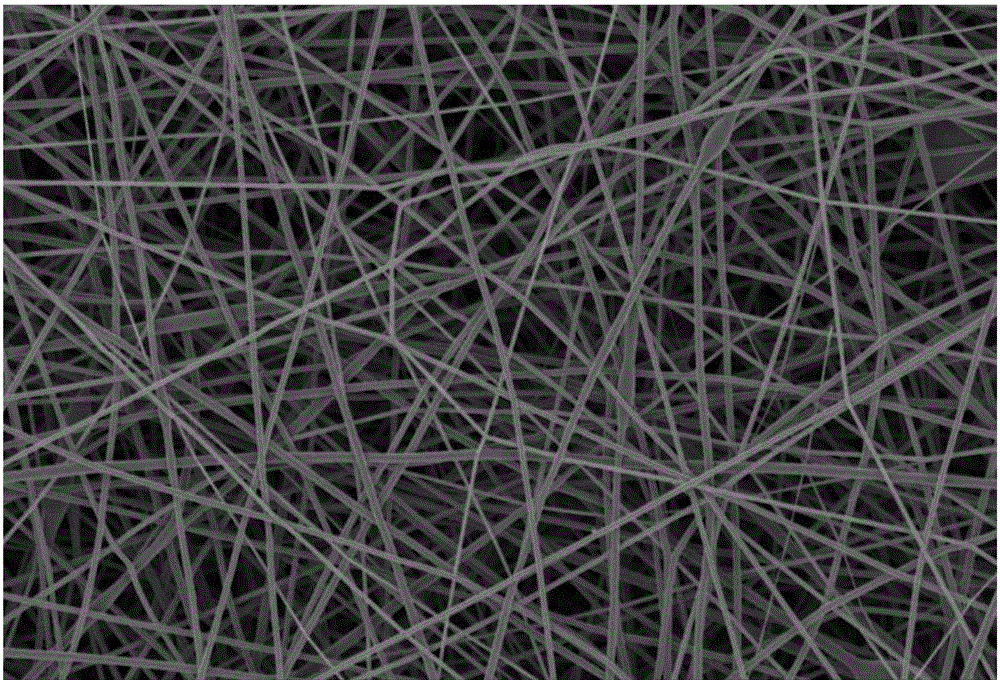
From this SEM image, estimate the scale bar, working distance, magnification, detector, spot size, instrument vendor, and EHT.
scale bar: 10000 nm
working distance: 24 mm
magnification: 2 K X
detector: SEI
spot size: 20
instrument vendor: JEOL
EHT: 5 kV